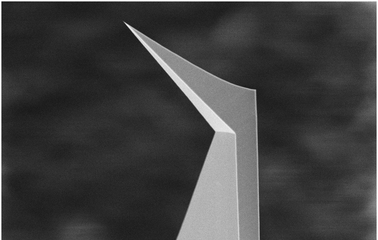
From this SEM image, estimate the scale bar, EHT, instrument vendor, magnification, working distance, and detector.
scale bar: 2000 nm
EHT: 5 kV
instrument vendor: Zeiss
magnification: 1.85 K X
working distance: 8.6 mm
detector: SE2